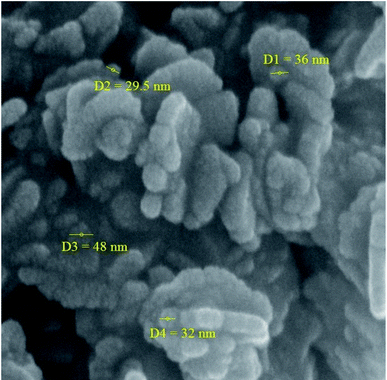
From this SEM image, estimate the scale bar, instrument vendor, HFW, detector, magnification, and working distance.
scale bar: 200 nm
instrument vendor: Tescan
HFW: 1.04 µm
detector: InBeam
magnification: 200 K X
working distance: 4.95 mm